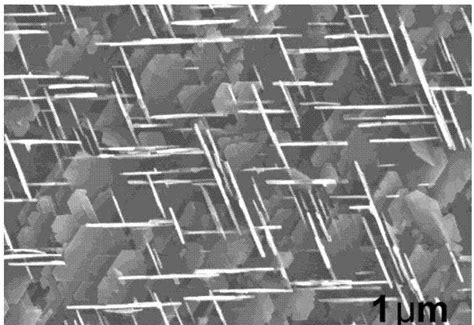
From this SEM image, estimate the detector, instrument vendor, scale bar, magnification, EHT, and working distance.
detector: SE(U)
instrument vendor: Hitachi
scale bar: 2000 nm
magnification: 20 K X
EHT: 15 kV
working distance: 11.1 mm